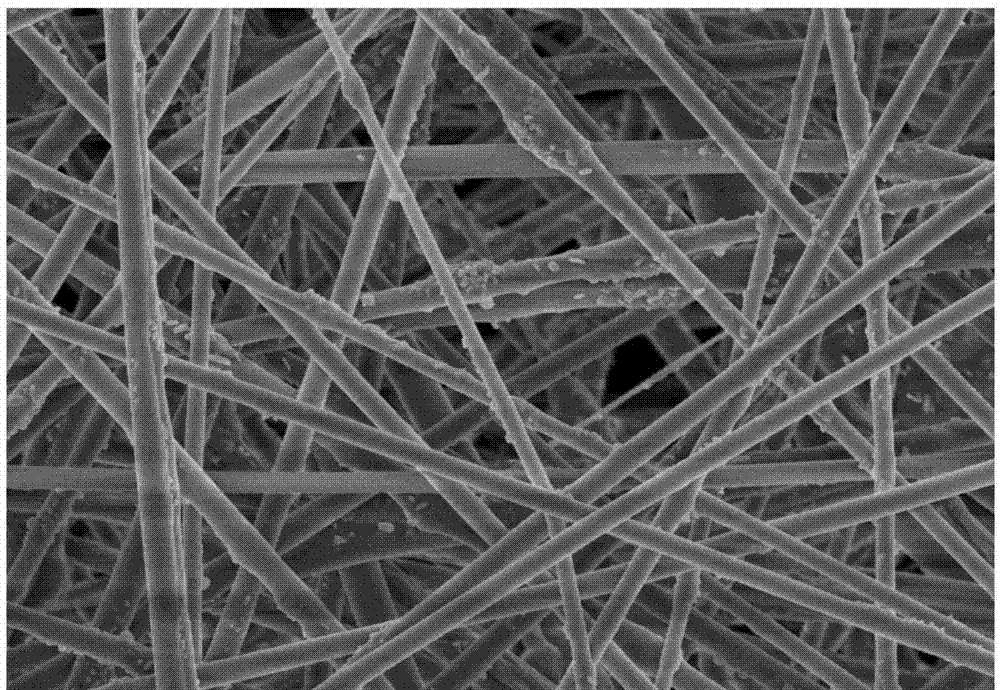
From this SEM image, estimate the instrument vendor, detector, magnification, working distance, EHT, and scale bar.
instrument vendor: Hitachi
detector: SE(U)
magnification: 8 K X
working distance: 6.7 mm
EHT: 3 kV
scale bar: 5000 nm